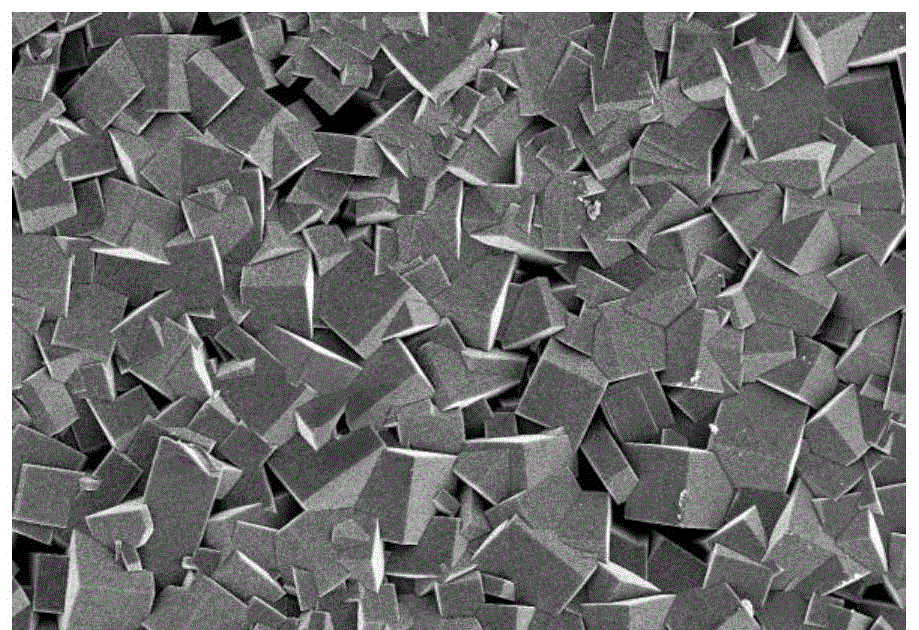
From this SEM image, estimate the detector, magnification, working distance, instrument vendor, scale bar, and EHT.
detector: SE(M)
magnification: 2 K X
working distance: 8.7 mm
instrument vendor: Hitachi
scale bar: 20000 nm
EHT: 5 kV